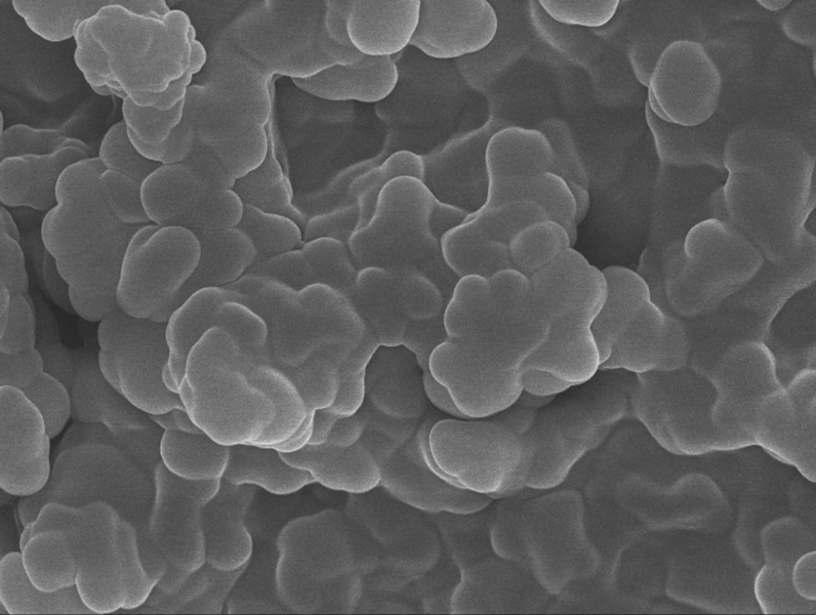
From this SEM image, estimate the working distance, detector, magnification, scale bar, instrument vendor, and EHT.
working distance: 8 mm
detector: SEI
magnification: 10 K X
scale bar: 1000 nm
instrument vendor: JEOL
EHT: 10 kV